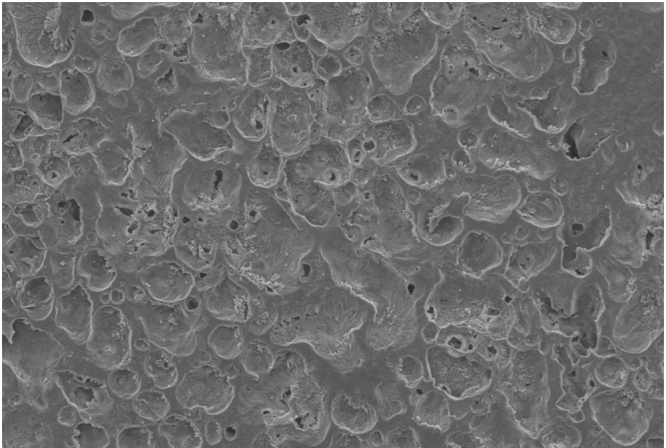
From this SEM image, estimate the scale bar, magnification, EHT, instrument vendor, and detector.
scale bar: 50000 nm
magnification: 0.5 K X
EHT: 6 kV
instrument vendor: JEOL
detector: SEI